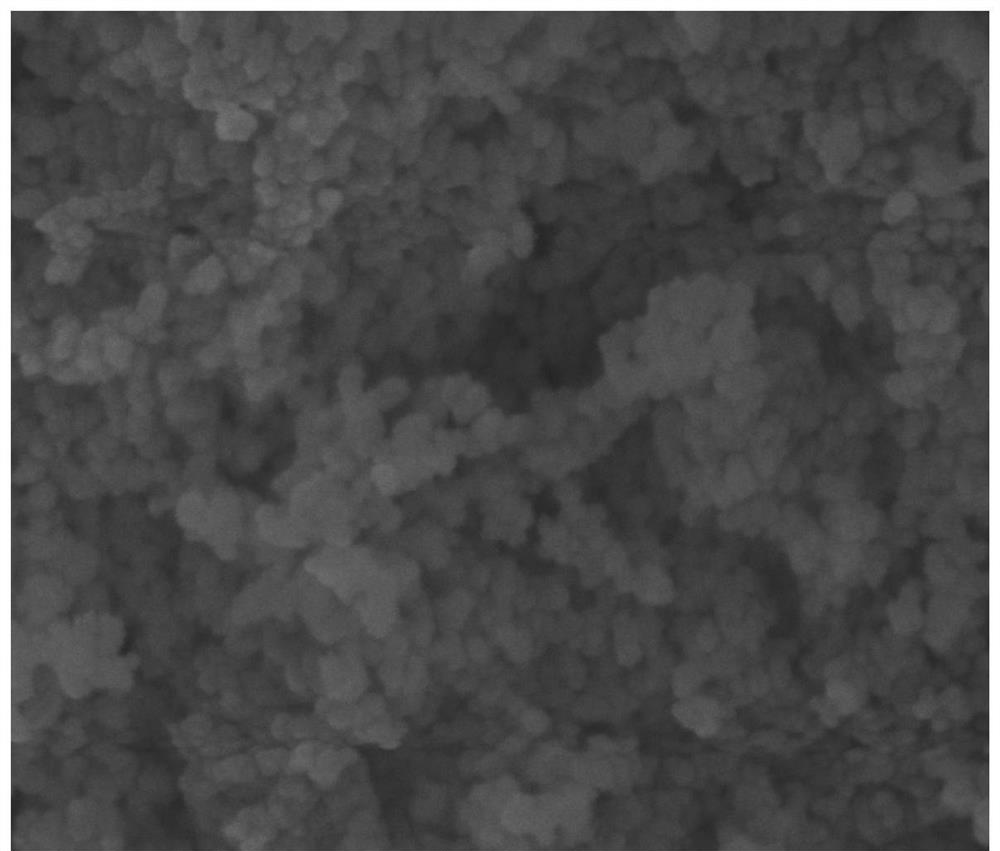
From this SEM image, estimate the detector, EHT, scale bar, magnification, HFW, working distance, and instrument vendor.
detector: ETD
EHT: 20 kV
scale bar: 1000 nm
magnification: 100 K X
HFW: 2.98 µm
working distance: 10.1 mm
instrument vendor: FEI